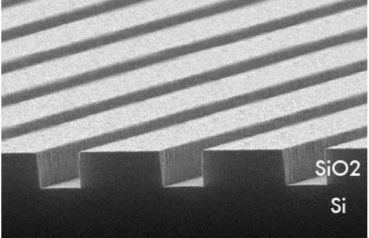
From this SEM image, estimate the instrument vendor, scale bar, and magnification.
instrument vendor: JEOL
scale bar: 2000 nm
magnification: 8 K X